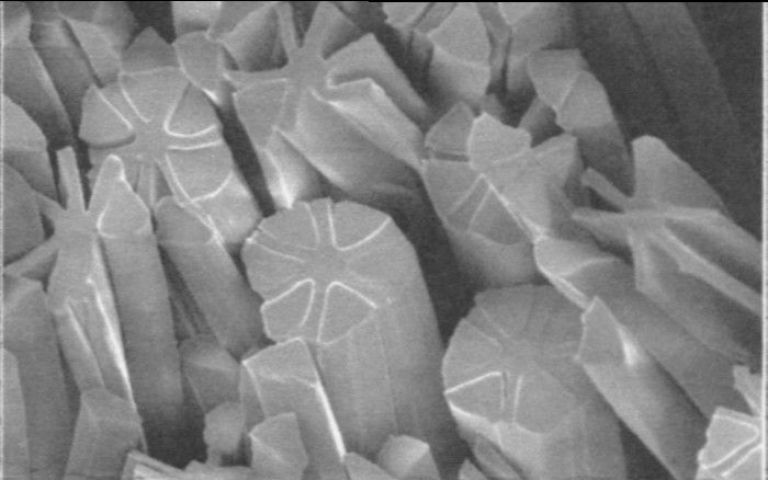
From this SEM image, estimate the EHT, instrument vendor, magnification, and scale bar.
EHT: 5 kV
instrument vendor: JEOL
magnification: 1.5 K X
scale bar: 10000 nm